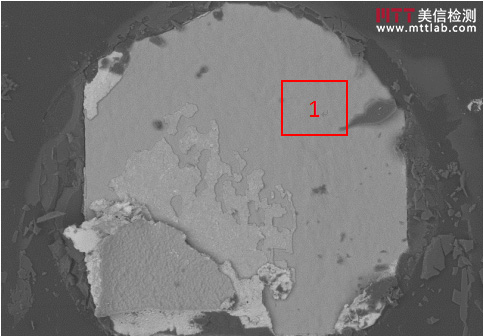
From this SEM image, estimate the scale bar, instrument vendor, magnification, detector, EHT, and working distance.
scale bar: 300000 nm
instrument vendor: Hitachi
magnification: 0.15 K X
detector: BSE3D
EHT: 15 kV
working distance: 10 mm